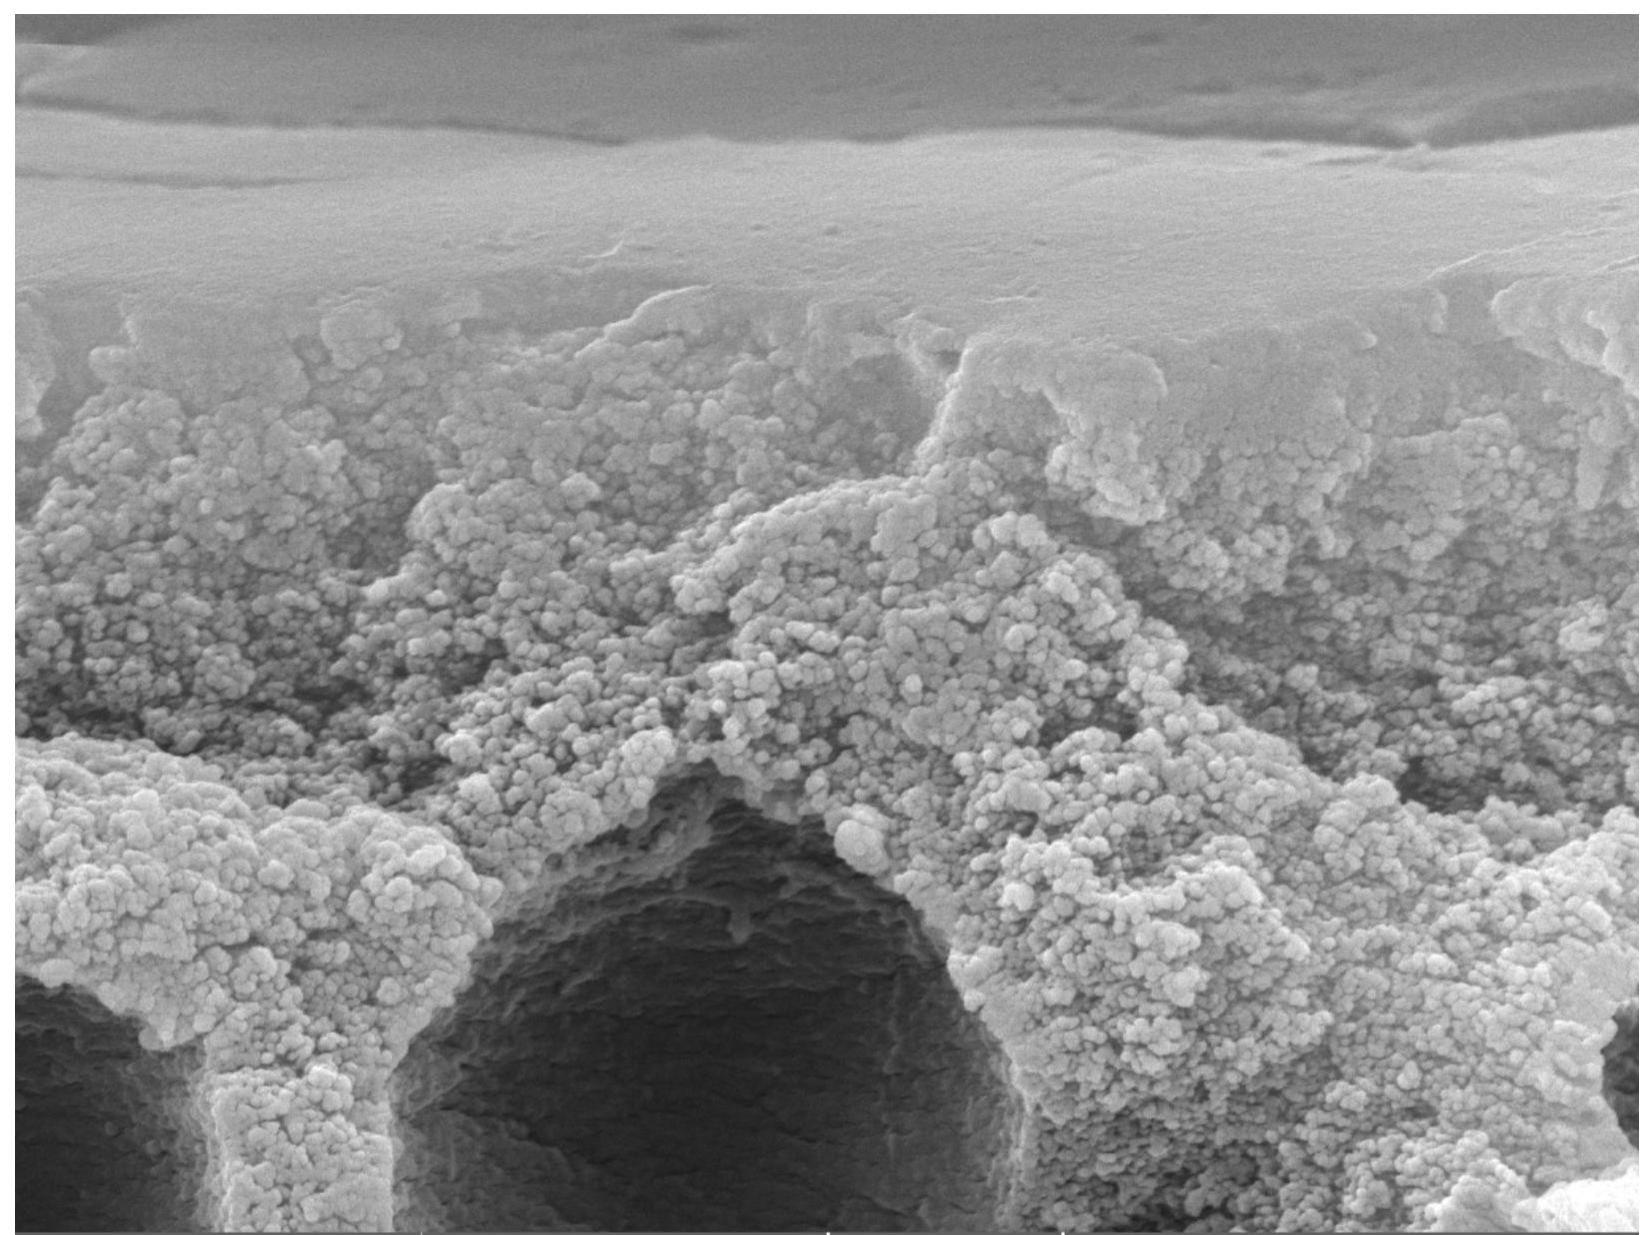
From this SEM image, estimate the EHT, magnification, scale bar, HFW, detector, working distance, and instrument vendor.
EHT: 15 kV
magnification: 20 K X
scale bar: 2000 nm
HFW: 13.8 µm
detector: SE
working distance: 9.04 mm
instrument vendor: Tescan